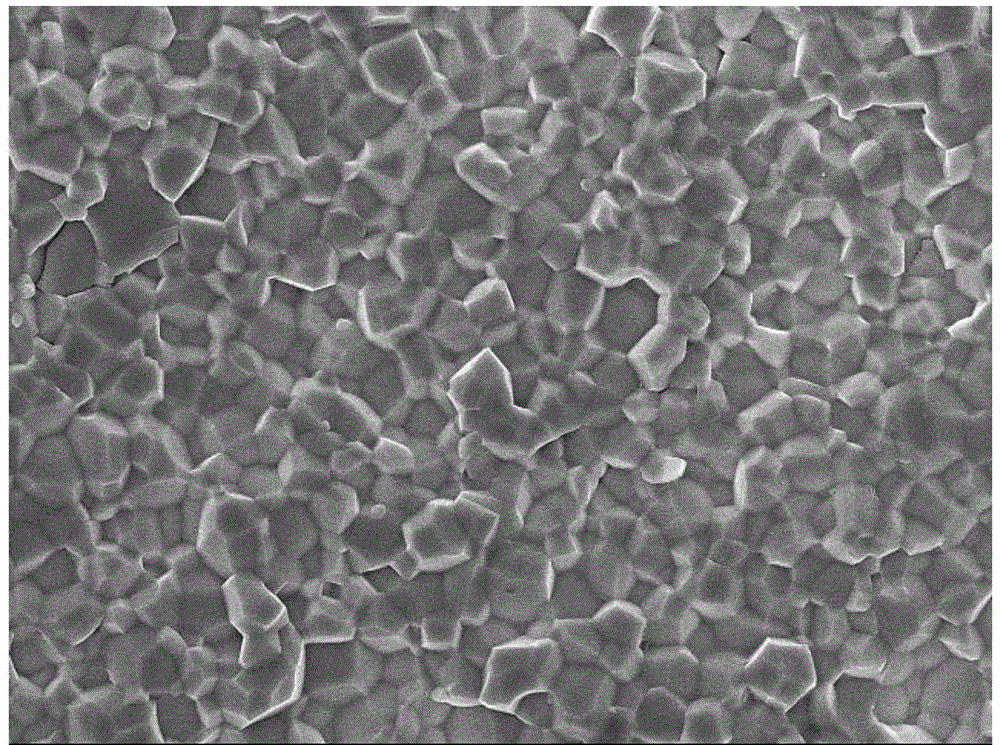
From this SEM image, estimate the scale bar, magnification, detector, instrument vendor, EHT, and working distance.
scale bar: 1000 nm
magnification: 5 K X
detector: SEI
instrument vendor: JEOL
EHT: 10 kV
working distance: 6 mm